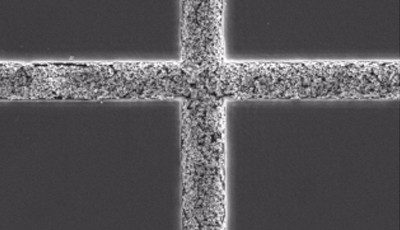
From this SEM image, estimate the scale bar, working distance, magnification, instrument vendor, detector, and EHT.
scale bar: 10000 nm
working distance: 10.16 mm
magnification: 5 K X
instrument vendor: Tescan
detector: SE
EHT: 10 kV